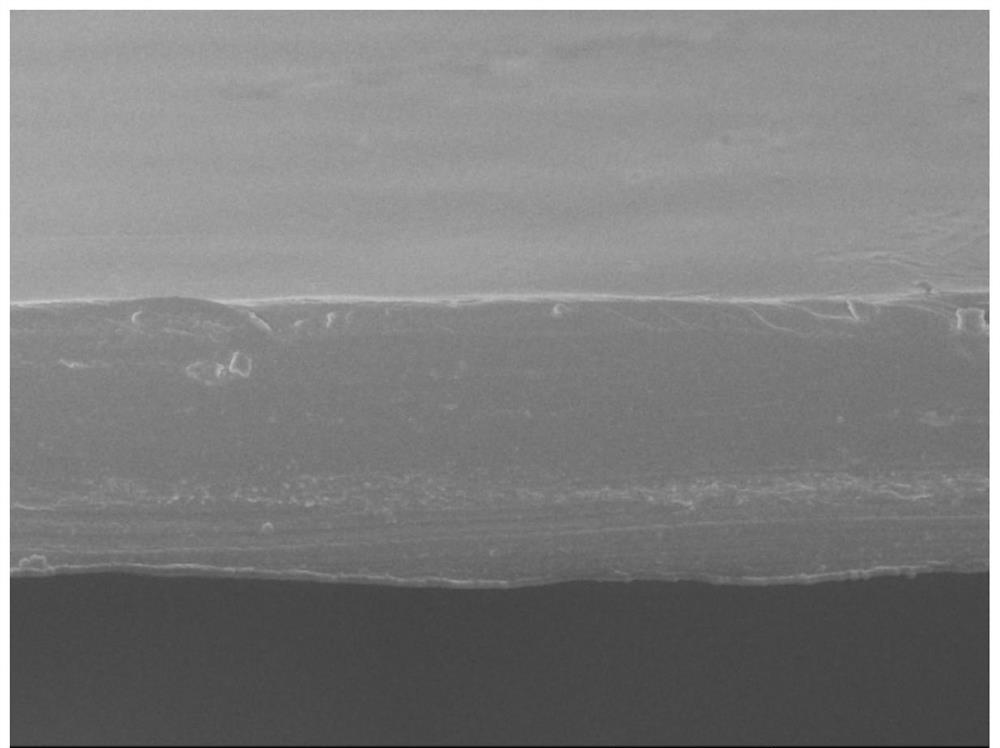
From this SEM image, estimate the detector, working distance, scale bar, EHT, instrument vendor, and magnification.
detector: SEI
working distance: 7.5 mm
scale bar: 10000 nm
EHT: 5 kV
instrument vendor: JEOL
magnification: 2 K X